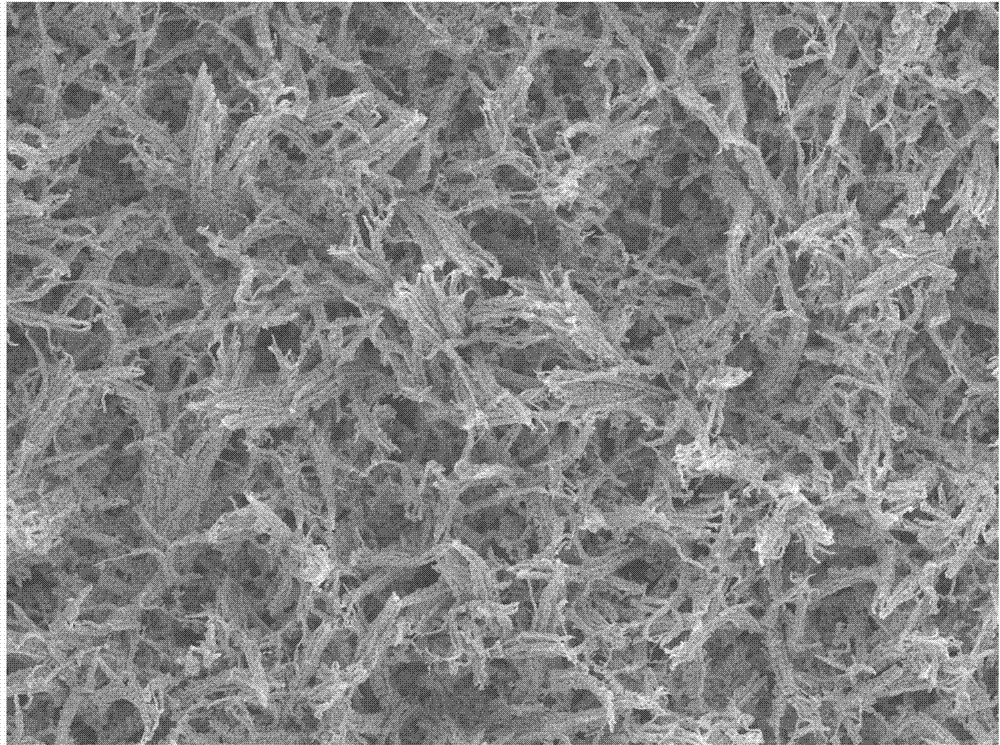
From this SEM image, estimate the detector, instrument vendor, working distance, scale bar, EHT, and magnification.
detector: SEI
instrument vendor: JEOL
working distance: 7.8 mm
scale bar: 10000 nm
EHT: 10 kV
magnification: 1 K X